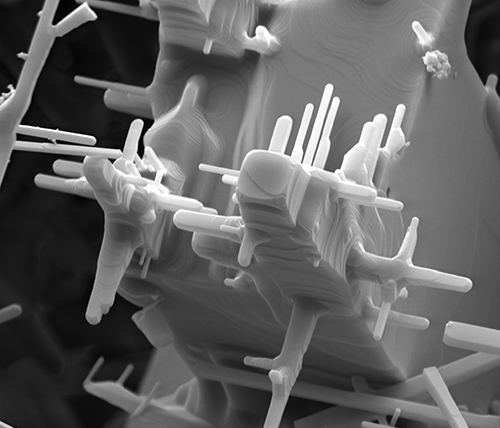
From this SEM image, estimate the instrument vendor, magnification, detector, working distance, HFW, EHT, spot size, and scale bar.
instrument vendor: FEI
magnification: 8.298 K X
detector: SE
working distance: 9 mm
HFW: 15.4 µm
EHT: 10 kV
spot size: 4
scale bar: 5000 nm